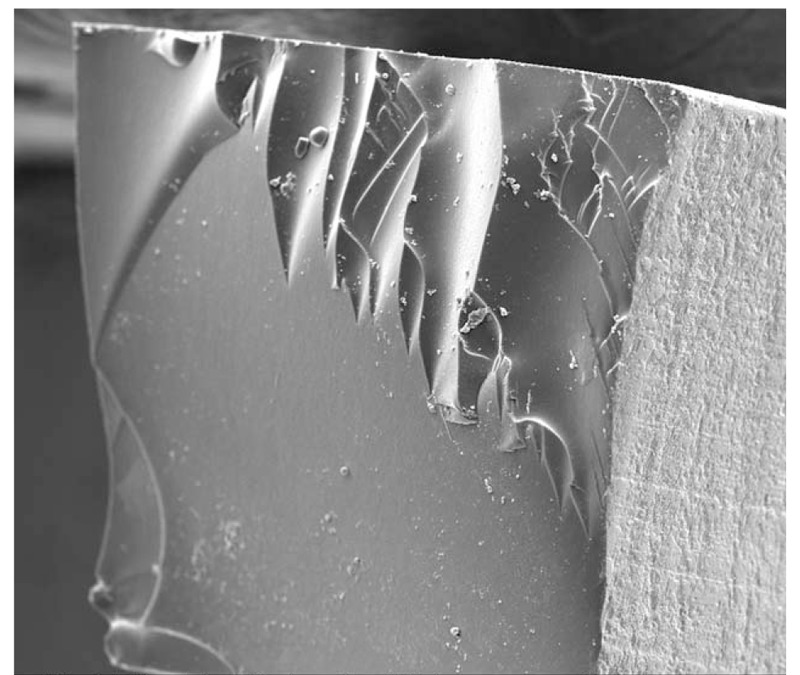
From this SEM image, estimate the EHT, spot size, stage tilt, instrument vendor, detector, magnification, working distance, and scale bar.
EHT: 10 kV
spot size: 3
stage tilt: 0°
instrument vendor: FEI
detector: ETD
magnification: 0.7 K X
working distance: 10.5 mm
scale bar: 100000 nm